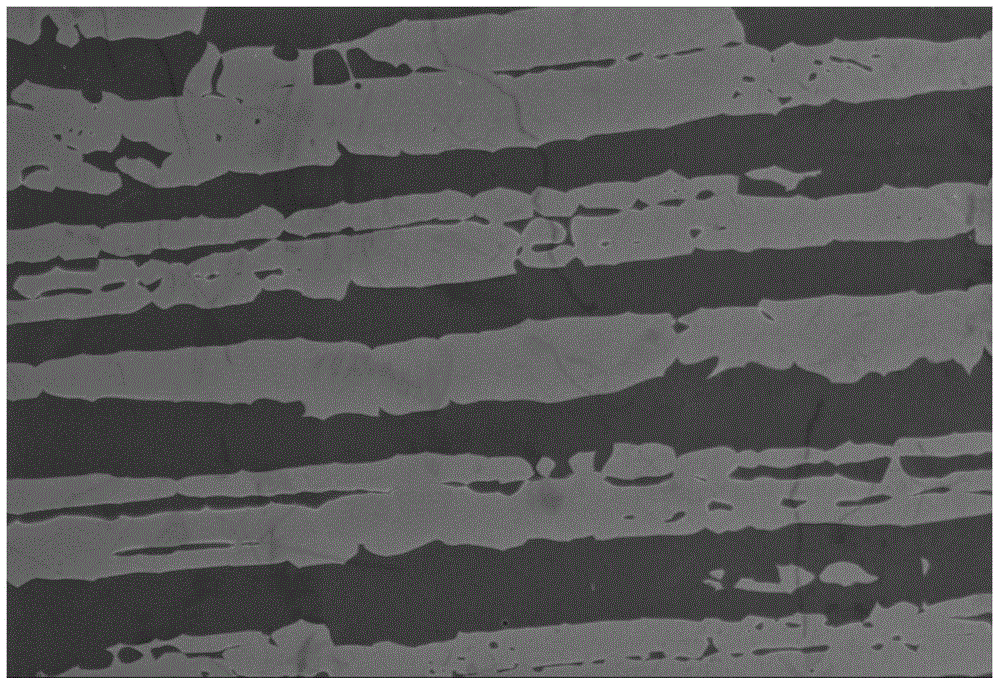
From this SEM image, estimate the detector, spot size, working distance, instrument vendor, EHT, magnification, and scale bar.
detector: SEI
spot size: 54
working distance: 10 mm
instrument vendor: JEOL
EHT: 20 kV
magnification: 0.5 K X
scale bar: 50000 nm